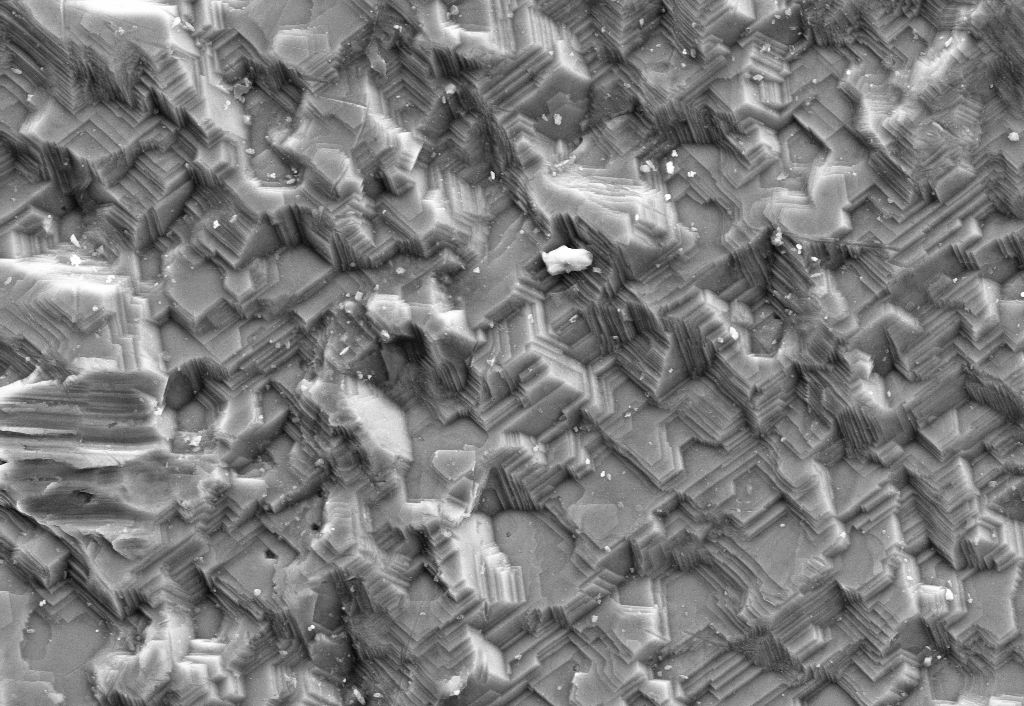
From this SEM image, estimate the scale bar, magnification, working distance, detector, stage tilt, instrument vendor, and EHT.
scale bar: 20000 nm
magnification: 1.5 K X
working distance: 11.8 mm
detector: SE2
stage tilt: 0°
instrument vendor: Zeiss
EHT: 15 kV